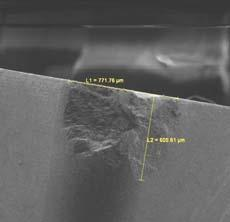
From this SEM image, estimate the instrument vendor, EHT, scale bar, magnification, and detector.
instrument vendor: Tescan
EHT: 5 kV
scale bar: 500000 nm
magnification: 0.135 K X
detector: SE Detector